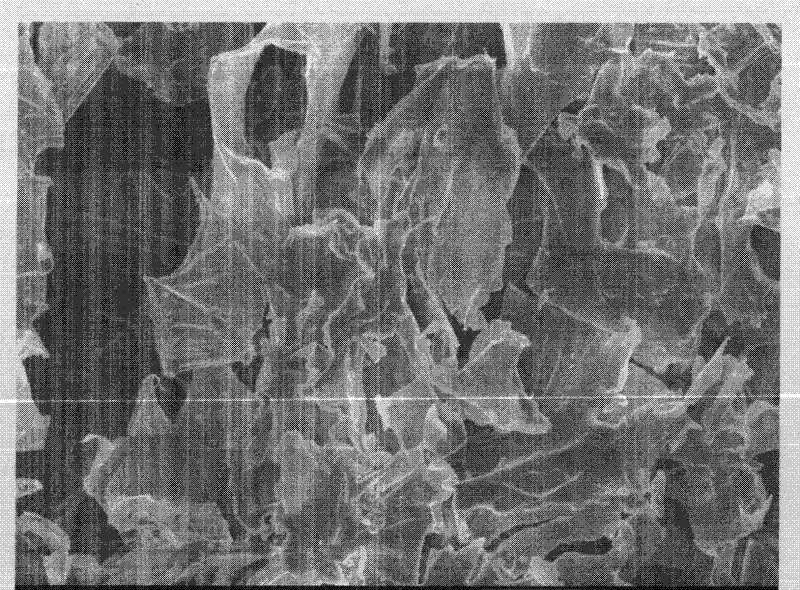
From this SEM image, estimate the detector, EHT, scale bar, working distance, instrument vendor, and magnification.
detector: SEI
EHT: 5 kV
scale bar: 10000 nm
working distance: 8.2 mm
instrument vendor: JEOL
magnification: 2 K X